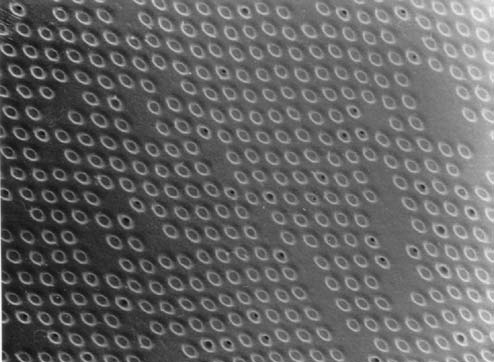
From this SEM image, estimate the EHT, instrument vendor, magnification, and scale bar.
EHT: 10 kV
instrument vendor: Hitachi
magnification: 20 K X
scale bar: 1500 nm